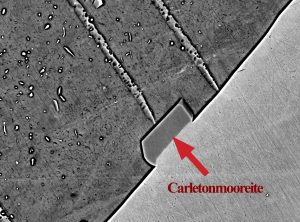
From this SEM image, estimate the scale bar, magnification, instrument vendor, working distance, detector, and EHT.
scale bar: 10000 nm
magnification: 1.7 K X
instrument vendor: JEOL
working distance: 10.8 mm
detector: BSE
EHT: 20 kV